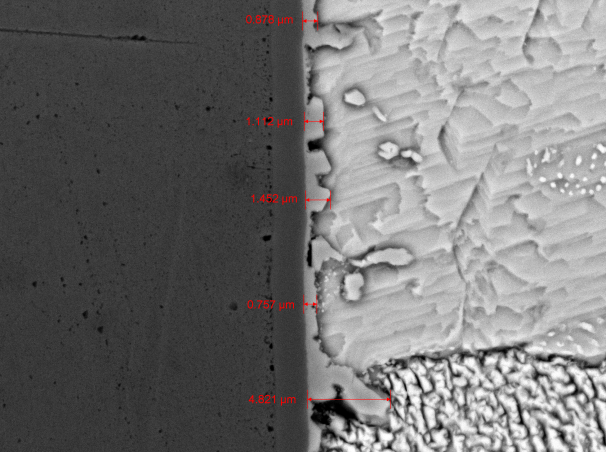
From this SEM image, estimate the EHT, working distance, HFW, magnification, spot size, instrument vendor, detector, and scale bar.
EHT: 20 kV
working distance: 7.62 mm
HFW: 35.13 µm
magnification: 10 K X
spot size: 1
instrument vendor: other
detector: BSE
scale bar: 2000 nm